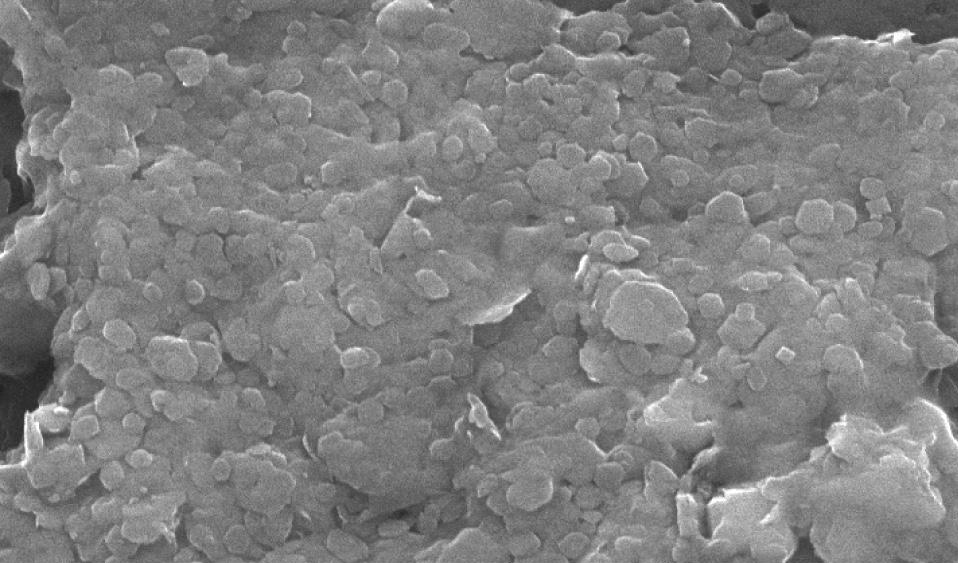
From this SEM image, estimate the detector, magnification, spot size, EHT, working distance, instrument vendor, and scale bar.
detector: SE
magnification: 20 K X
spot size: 3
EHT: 20 kV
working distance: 10 mm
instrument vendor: FEI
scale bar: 1000 nm